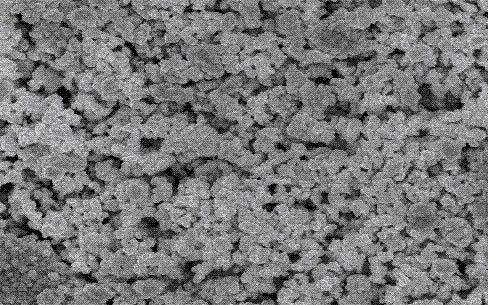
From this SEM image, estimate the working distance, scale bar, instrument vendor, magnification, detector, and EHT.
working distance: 8 mm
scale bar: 1000 nm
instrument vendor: JEOL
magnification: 10 K X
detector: SEI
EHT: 15 kV